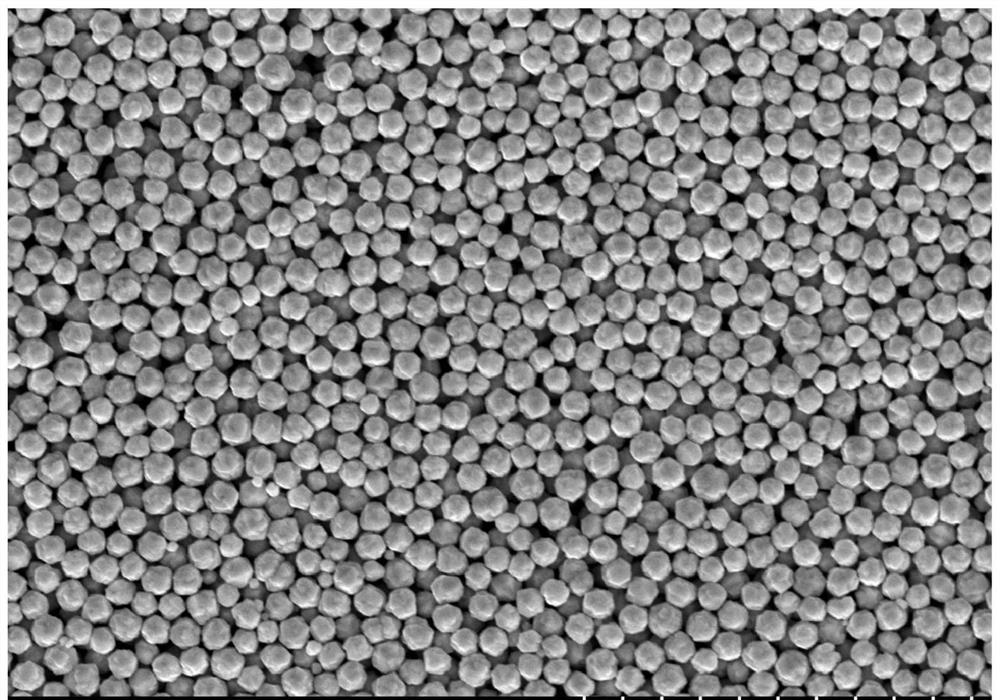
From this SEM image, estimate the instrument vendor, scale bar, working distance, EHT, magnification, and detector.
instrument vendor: Hitachi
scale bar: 1000 nm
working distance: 7.5 mm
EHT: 3 kV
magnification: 50 K X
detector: SE(M)